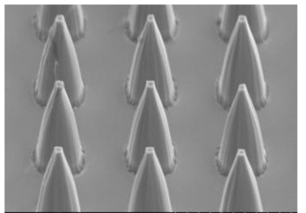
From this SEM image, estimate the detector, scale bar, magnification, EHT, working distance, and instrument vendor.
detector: SE(M)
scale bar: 5000 nm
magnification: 10 K X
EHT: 5 kV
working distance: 10.5 mm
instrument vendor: Hitachi